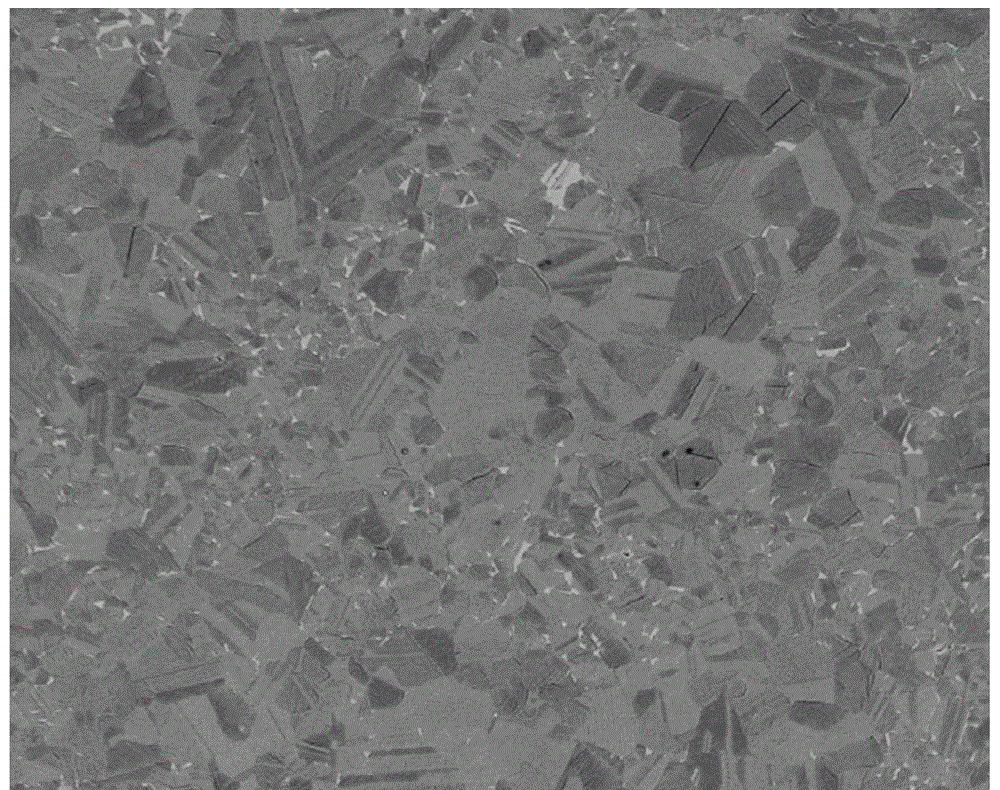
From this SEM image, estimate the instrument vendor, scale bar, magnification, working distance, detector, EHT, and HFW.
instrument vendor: FEI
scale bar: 100000 nm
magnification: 0.5 K X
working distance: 6.9 mm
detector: BSED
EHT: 18 kV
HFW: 480 µm